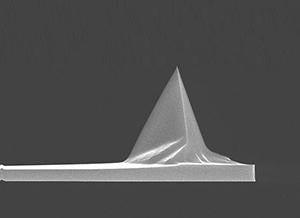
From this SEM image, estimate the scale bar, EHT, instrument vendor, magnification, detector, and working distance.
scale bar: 10000 nm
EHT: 15 kV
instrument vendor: FEI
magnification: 3.5 K X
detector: TLD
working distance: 4.4 mm